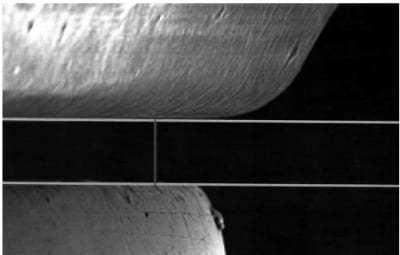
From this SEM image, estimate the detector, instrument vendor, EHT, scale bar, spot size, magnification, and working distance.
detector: SE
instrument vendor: FEI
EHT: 20 kV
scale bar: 100000 nm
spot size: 4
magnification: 0.25 K X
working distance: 16.8 mm